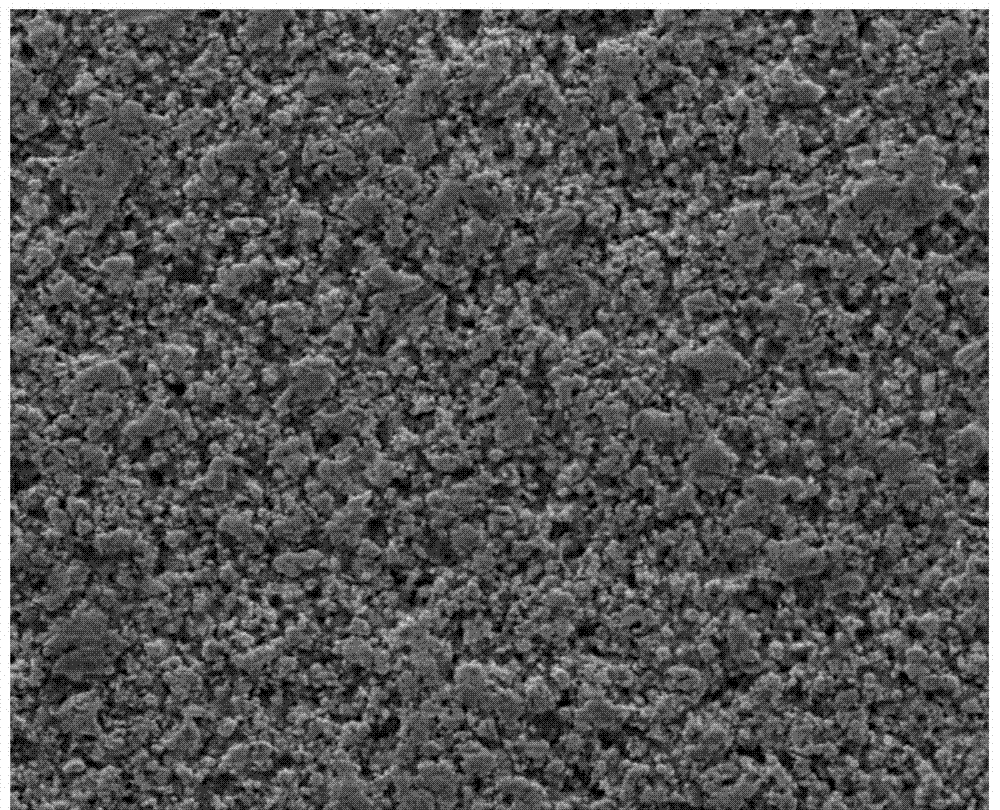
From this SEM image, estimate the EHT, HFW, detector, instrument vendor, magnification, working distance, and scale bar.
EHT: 20 kV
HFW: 160 µm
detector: ETD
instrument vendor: FEI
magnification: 1.6 K X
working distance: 10.8 mm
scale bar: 50000 nm